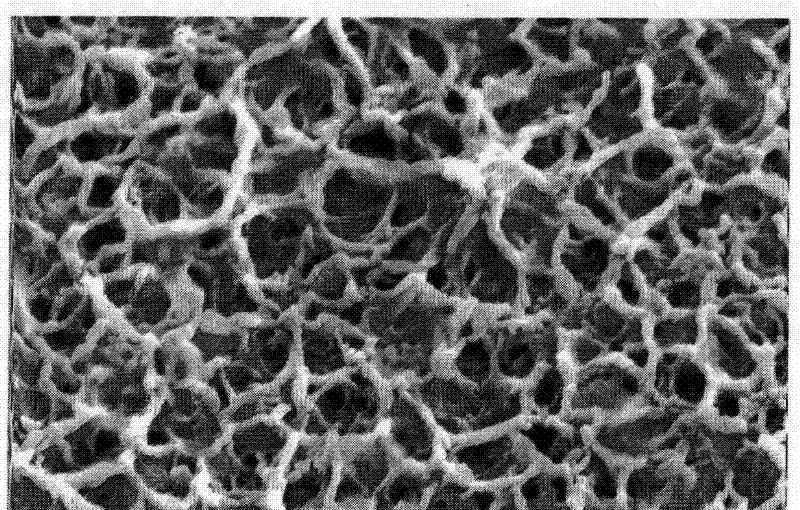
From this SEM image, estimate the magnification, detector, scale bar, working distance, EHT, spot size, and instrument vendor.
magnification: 30 K X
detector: SE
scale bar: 2000 nm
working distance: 6.4 mm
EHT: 20 kV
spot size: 3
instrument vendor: FEI